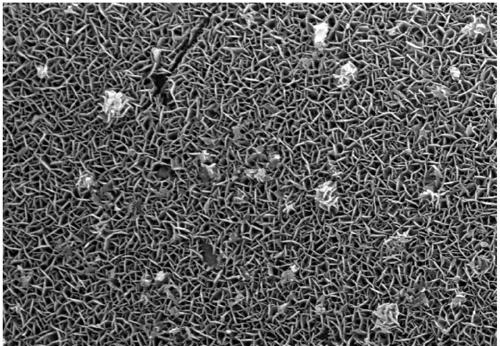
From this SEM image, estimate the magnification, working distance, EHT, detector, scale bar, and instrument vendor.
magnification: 18 K X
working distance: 5.7 mm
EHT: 10 kV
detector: SE(L)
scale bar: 3000 nm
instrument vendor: Hitachi